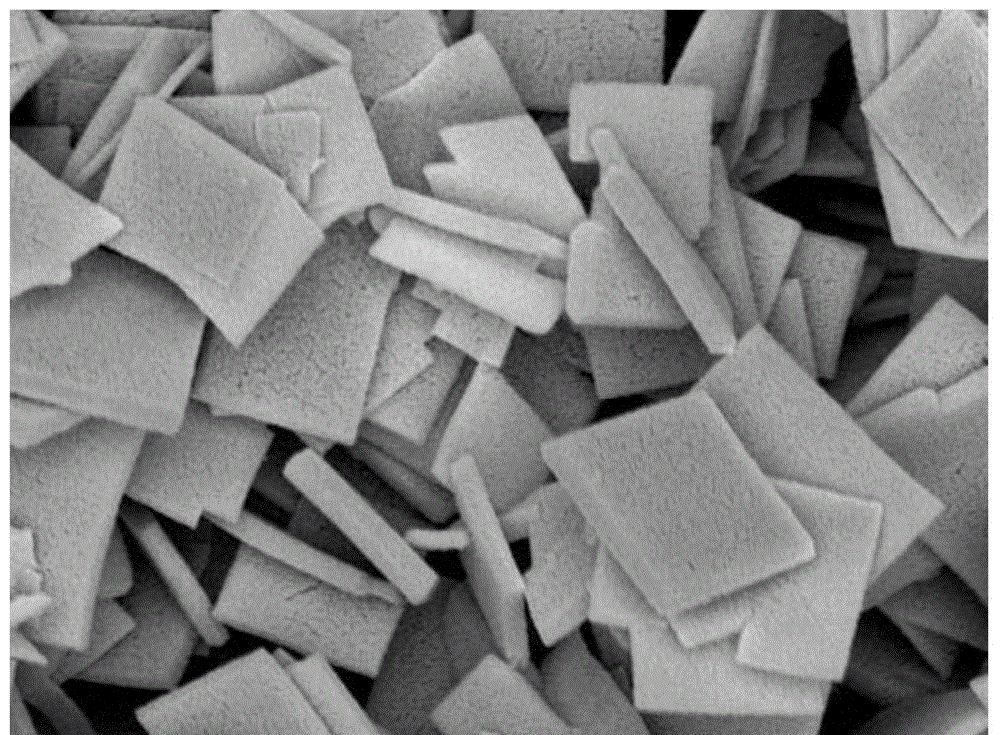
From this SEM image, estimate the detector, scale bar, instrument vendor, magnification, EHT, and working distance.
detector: SEI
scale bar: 1000 nm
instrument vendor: JEOL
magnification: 7 K X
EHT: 8 kV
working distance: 7.7 mm